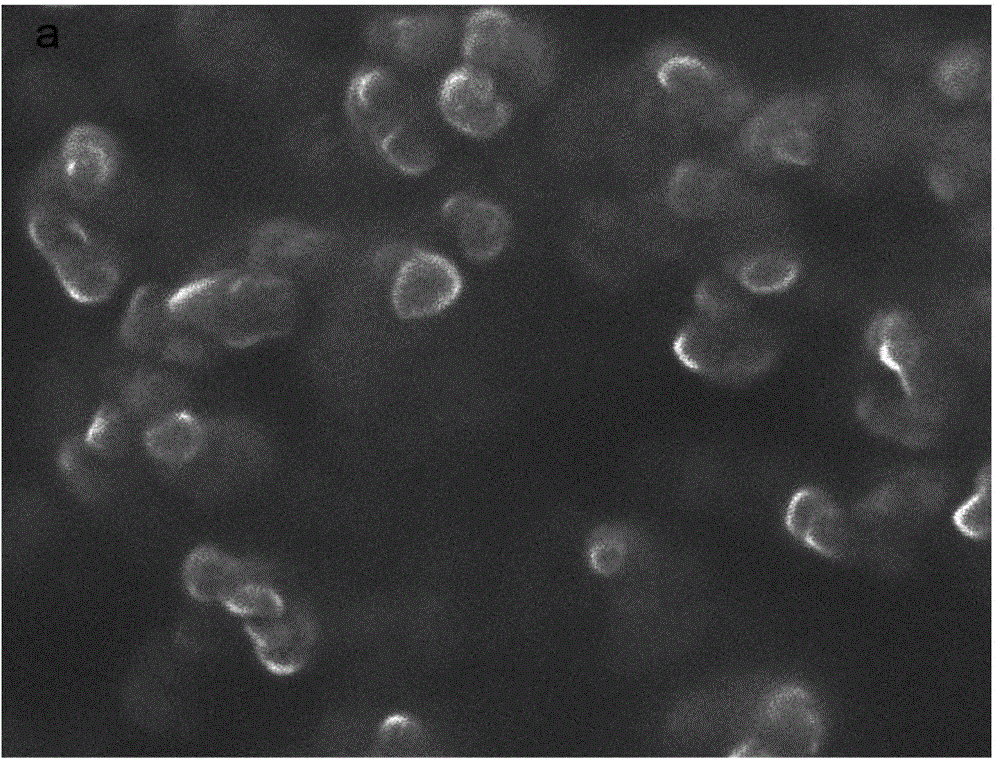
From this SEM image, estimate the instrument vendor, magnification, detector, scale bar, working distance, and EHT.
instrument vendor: FEI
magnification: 300 K X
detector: SE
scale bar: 400 nm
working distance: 8.7 mm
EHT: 10 kV